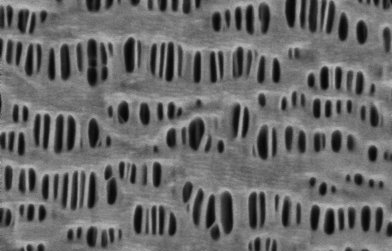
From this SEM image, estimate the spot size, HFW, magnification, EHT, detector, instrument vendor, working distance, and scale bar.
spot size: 6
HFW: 2.54 µm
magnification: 50 K X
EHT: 0.5 kV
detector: T1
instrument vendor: Thermo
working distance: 3.5986 mm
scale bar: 2540 nm